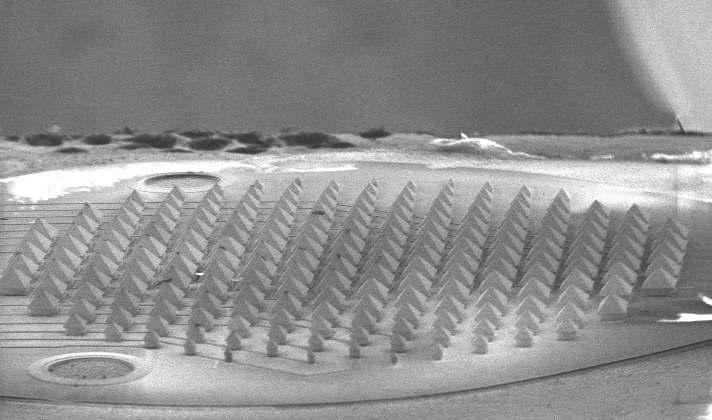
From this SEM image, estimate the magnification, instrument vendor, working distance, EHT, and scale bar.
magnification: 0.075 K X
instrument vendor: FEI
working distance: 18.5 mm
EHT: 5 kV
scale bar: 500000 nm